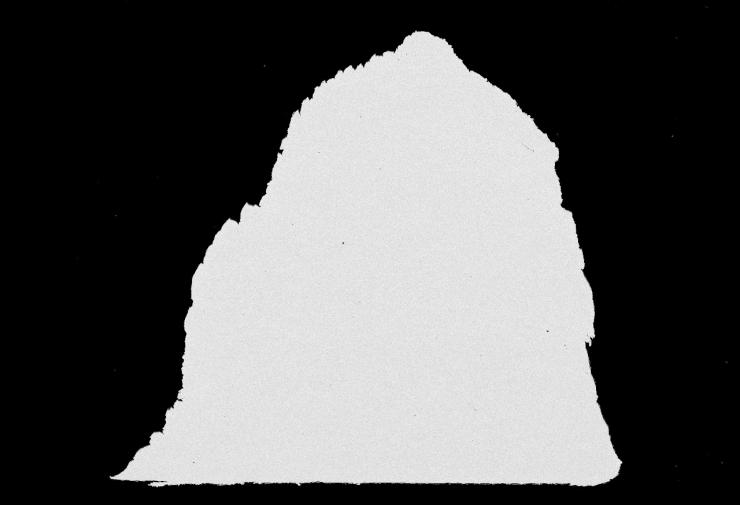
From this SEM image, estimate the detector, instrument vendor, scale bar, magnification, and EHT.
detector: BSE-COMP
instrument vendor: Hitachi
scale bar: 500000 nm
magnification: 0.1 K X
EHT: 15 kV